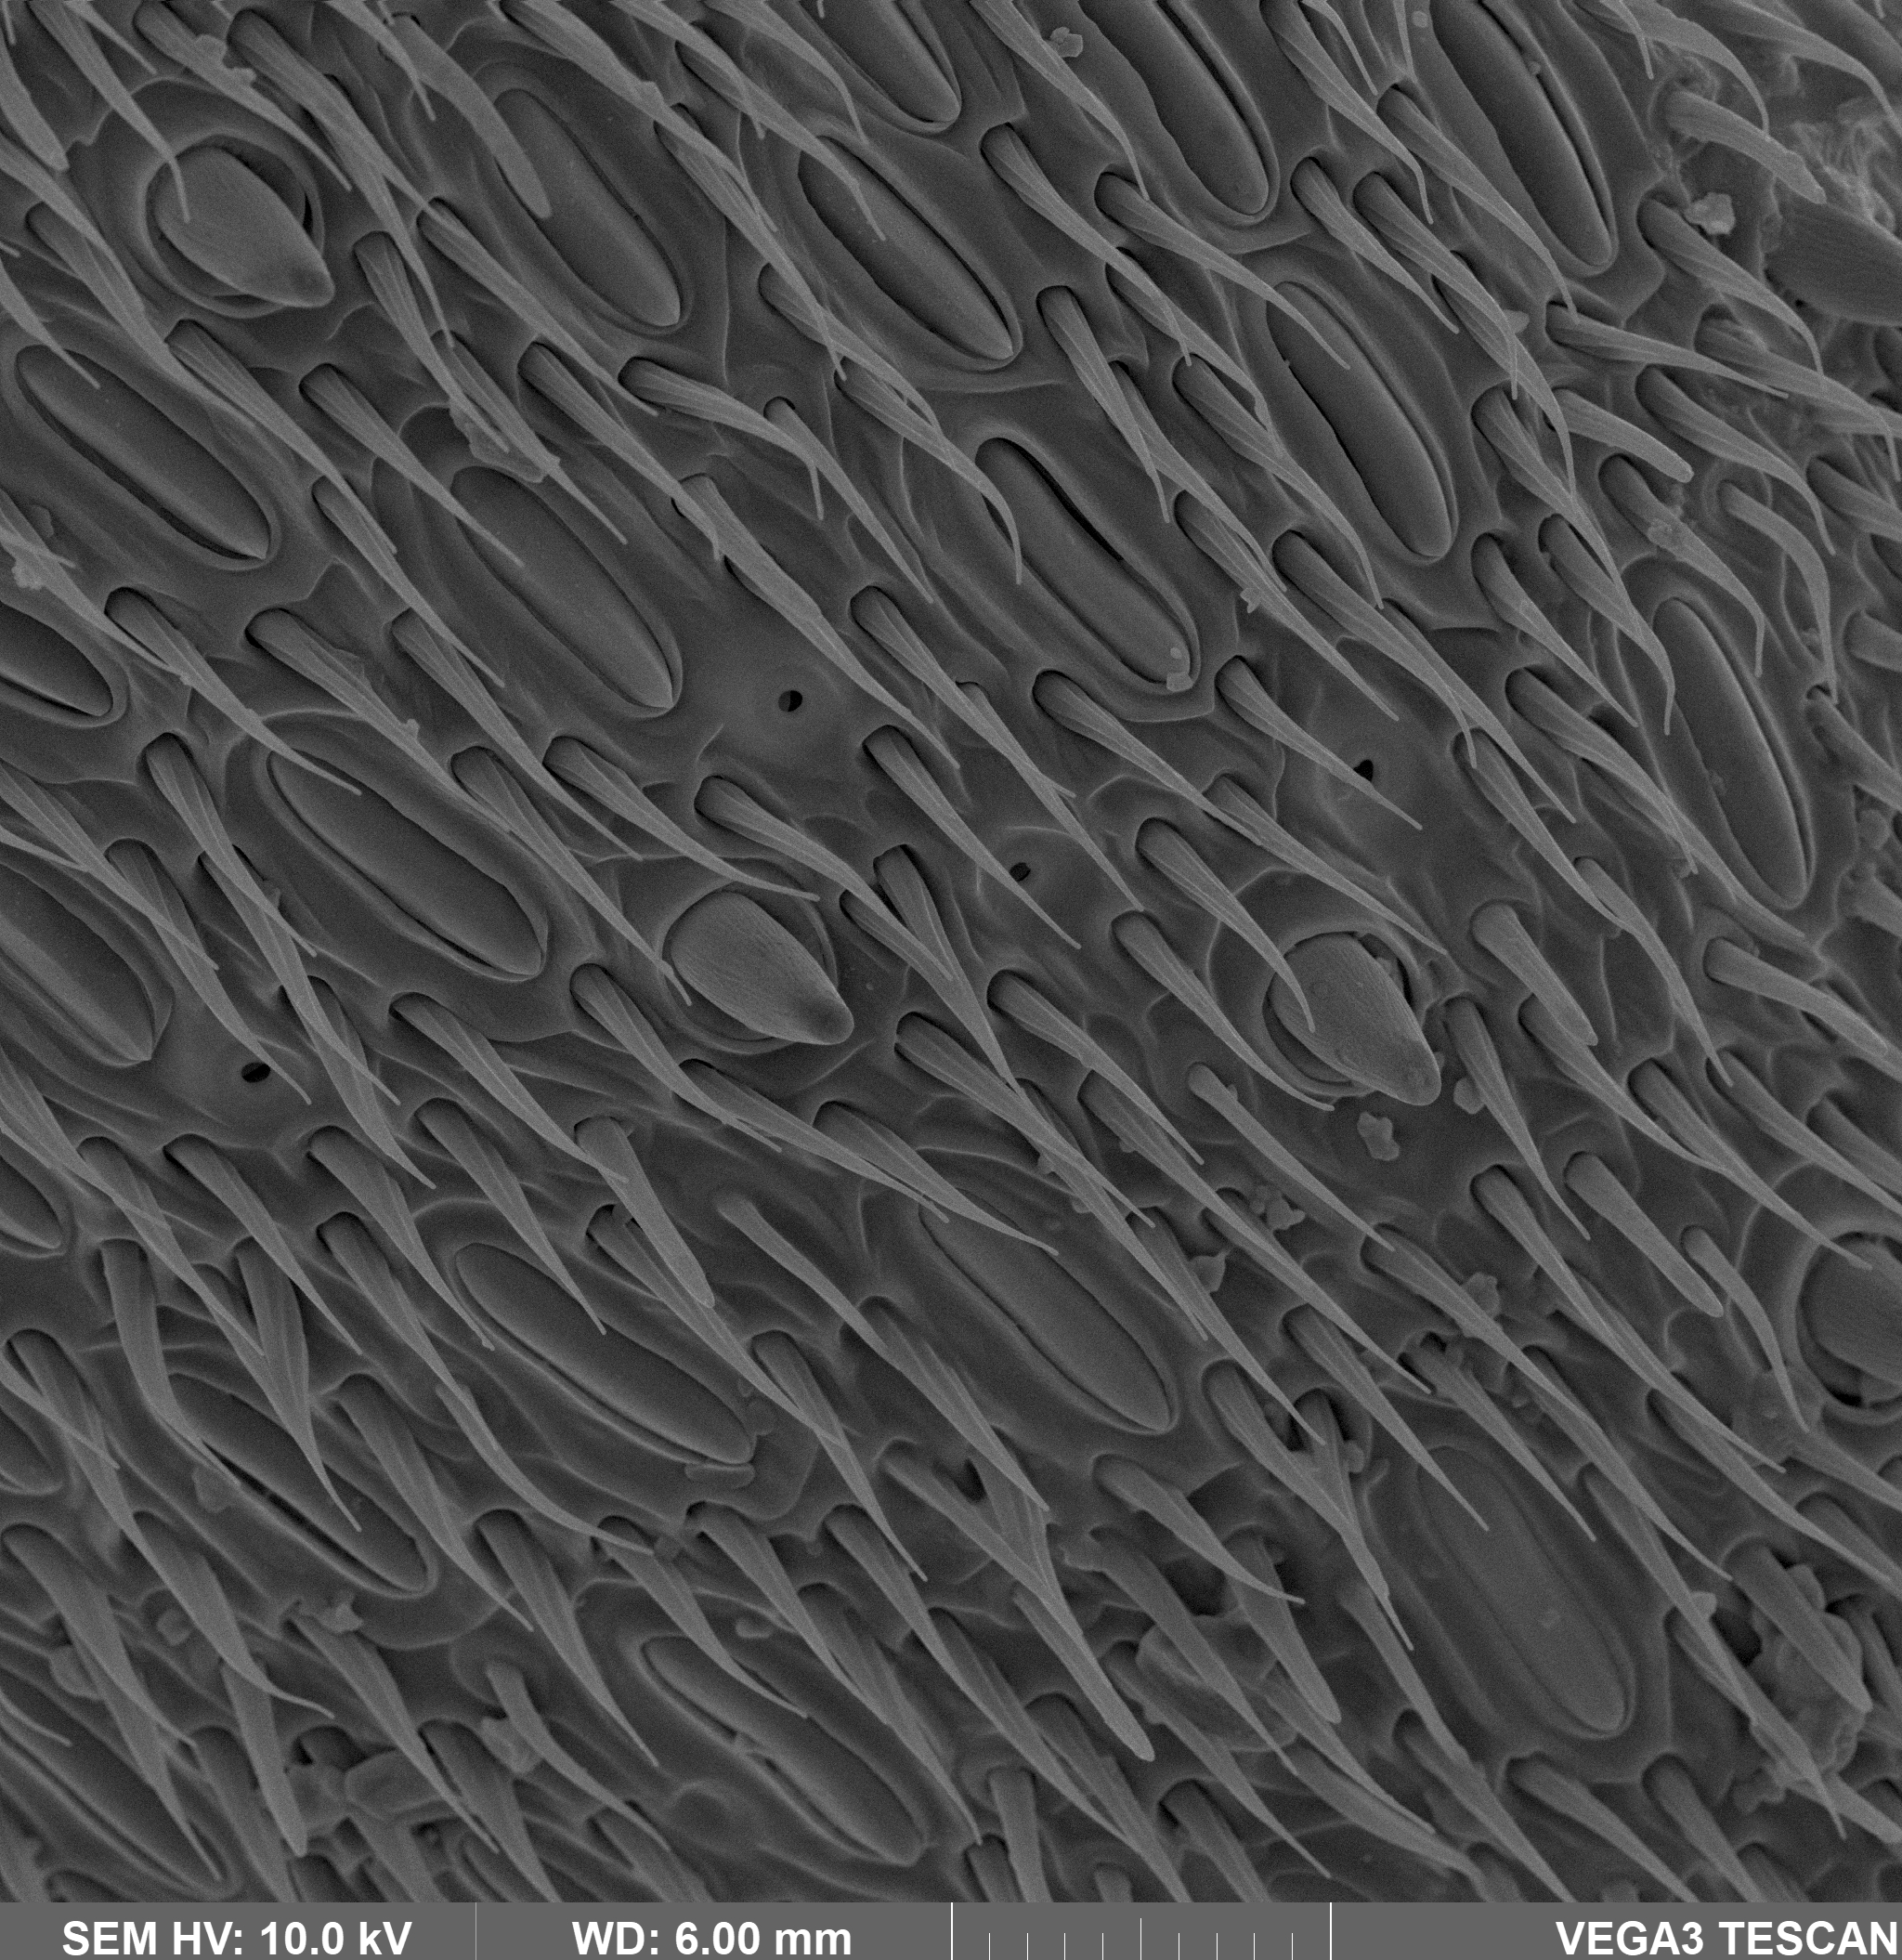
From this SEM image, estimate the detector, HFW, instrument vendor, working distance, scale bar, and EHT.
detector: SE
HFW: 100 µm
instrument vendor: Tescan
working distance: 6 mm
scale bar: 20000 nm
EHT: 10 kV